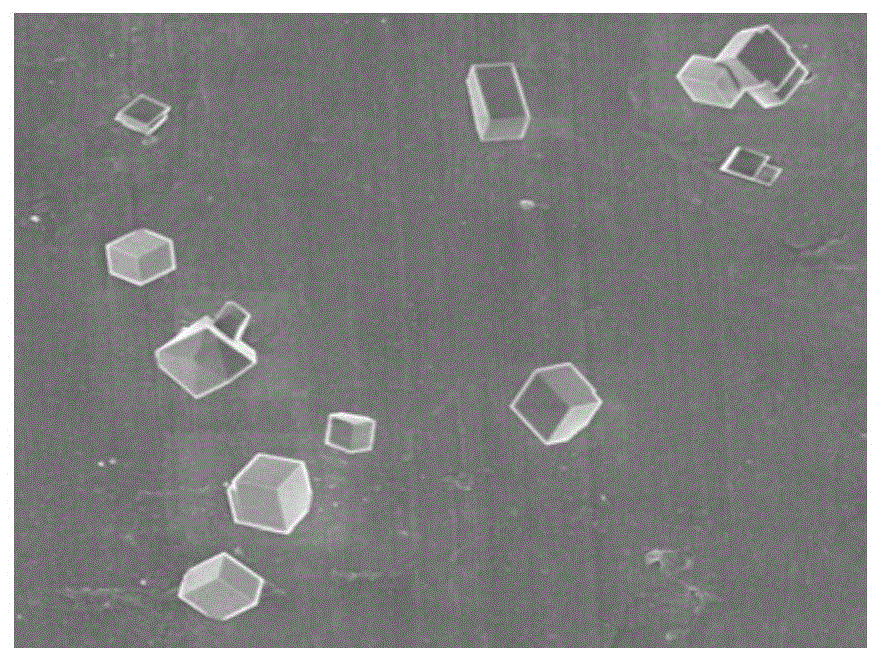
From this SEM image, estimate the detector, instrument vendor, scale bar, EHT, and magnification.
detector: SEI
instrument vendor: JEOL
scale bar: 1000 nm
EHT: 2 kV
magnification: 10 K X